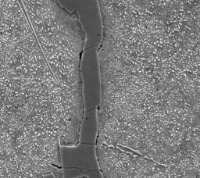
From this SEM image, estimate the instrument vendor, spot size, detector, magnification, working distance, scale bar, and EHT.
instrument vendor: FEI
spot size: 5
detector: SE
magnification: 1.964 K X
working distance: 10 mm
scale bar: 10000 nm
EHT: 20 kV